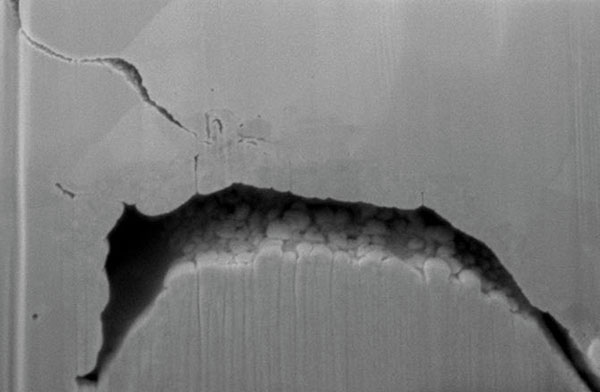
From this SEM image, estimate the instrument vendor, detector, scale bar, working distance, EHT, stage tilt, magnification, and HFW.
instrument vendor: FEI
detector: SE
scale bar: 1000 nm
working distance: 4 mm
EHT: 2 kV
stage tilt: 52°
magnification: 60 K X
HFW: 3.45 µm